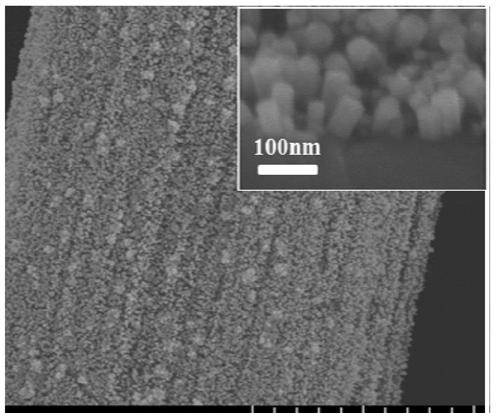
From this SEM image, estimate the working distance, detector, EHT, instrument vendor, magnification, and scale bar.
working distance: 11.2 mm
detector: SE(UL)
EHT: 5 kV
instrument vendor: Hitachi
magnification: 13 K X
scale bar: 4000 nm